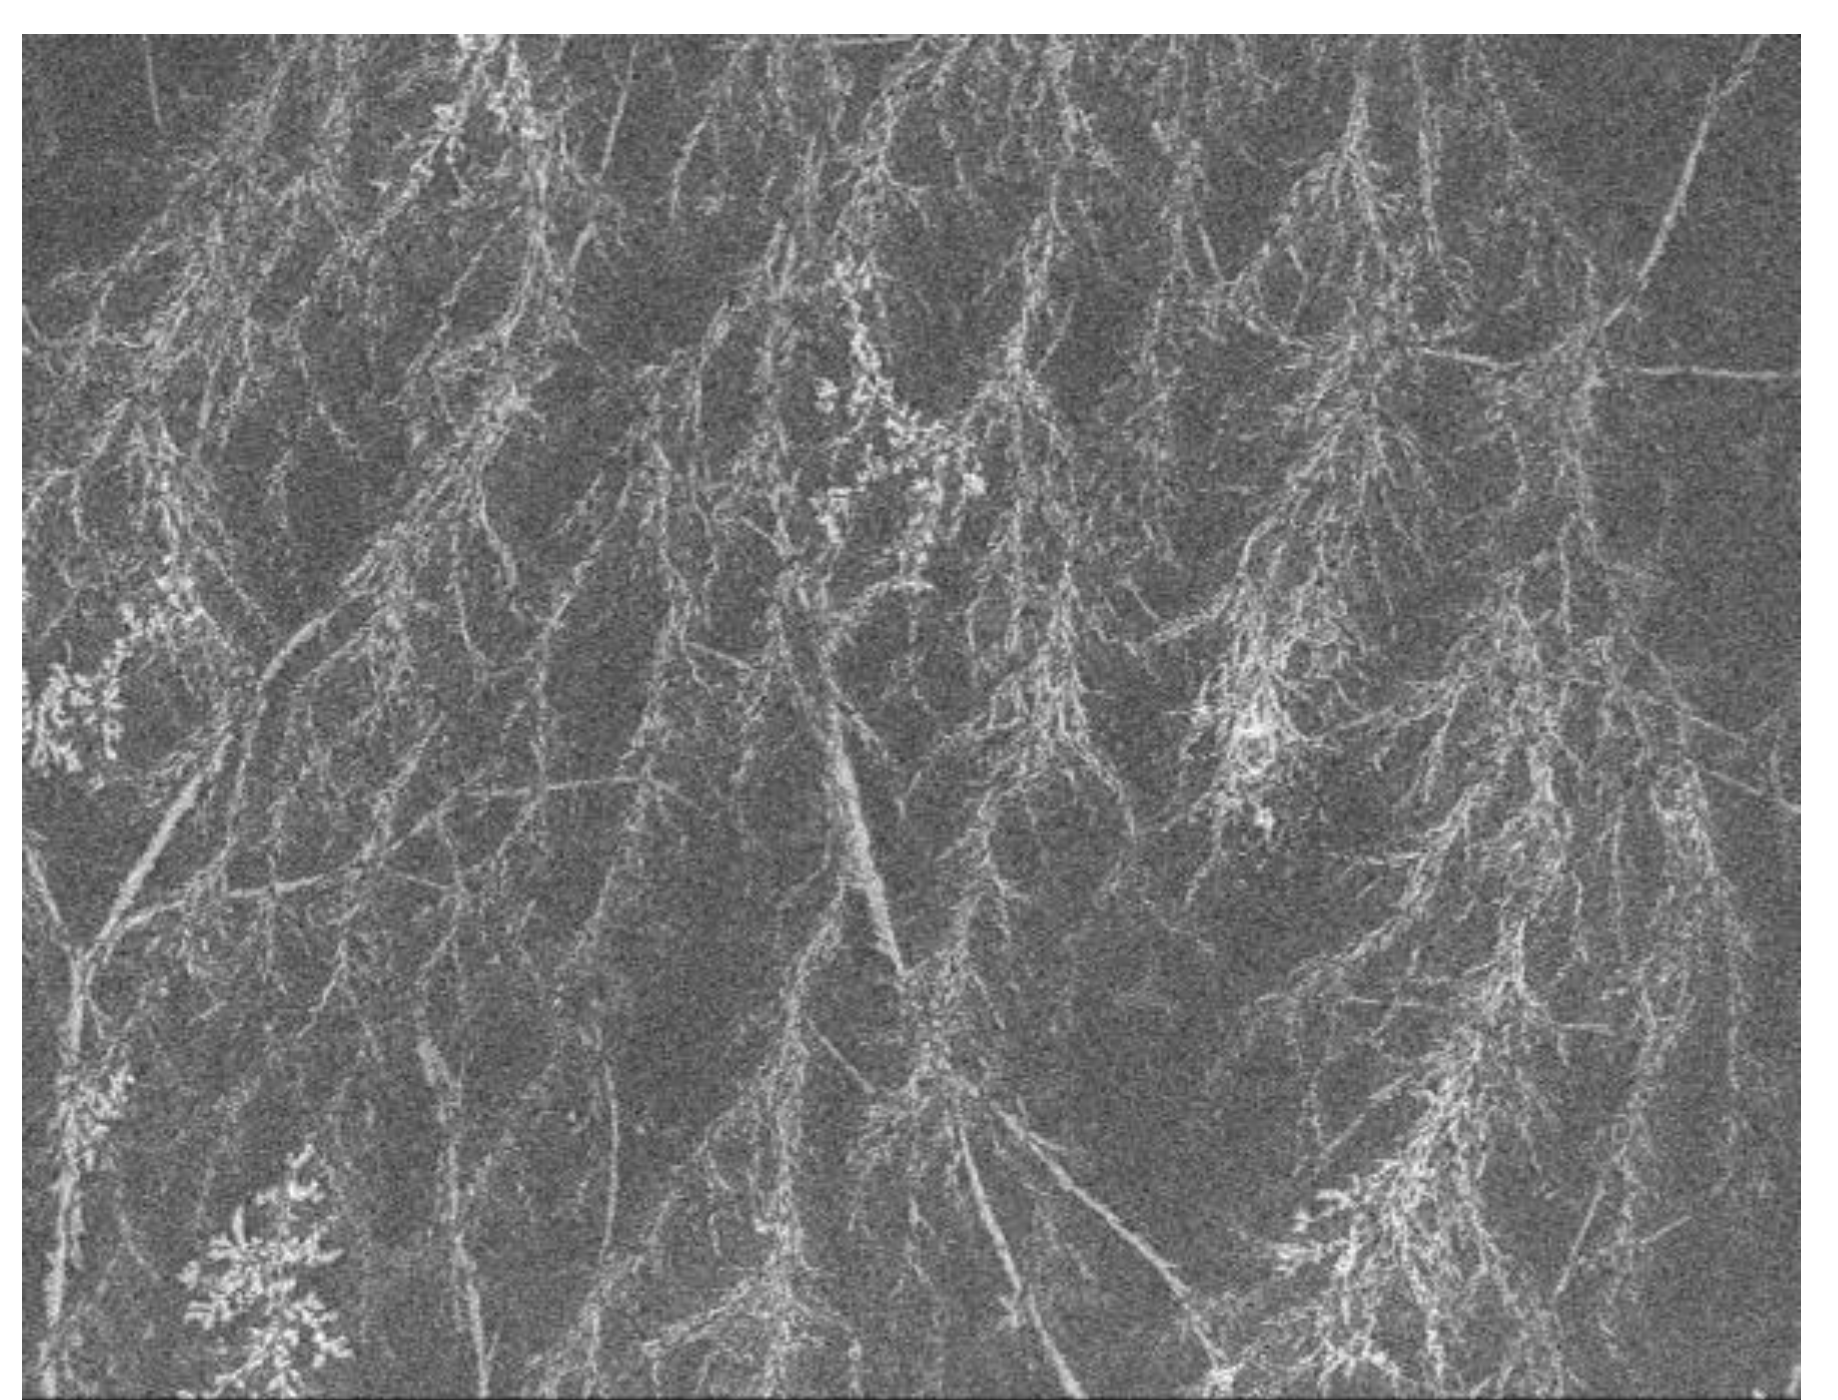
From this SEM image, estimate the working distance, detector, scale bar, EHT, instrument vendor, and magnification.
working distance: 7.2 mm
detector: SEI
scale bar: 10000 nm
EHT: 3 kV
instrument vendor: JEOL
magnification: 1 K X